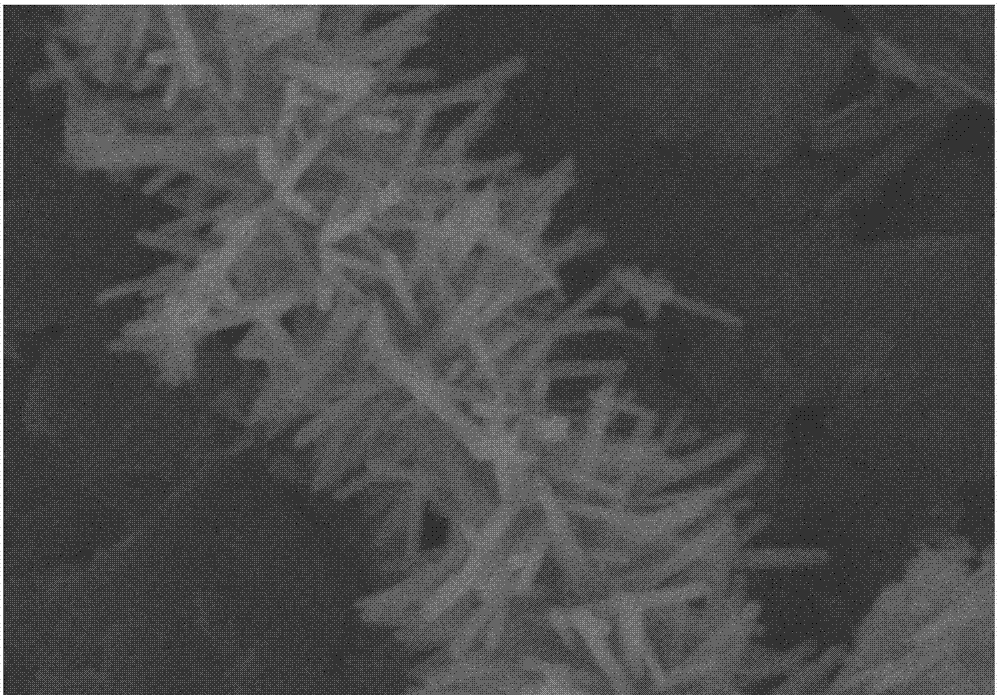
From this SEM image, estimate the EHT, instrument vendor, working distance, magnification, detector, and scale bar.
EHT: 5 kV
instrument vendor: Hitachi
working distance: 10.7 mm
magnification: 50 K X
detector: SE(M)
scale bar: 1000 nm